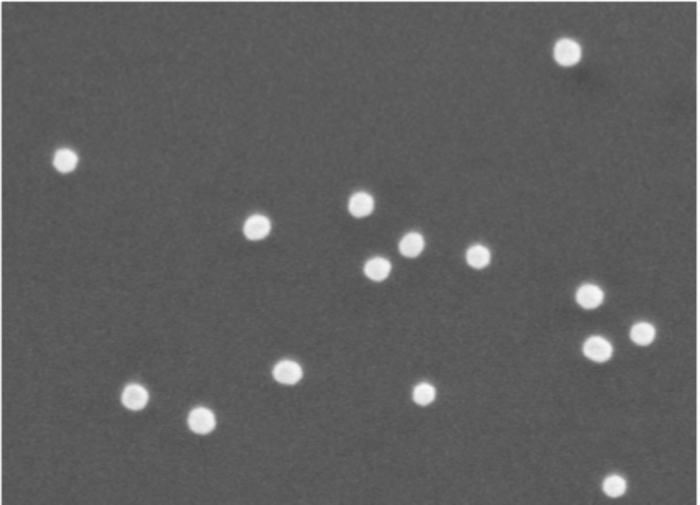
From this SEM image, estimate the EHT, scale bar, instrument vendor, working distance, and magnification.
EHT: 5 kV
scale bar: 100 nm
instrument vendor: JEOL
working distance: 15 mm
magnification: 45 K X